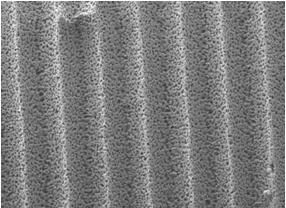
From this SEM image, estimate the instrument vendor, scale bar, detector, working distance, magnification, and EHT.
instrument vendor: JEOL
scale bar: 10000 nm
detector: LEI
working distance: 8 mm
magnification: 0.8 K X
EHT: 10 kV